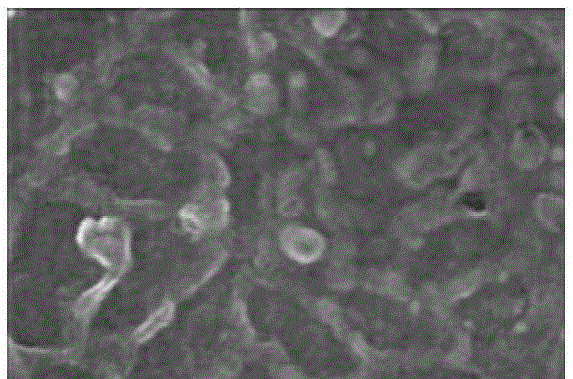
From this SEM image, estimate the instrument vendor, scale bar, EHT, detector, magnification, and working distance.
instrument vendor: Hitachi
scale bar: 500 nm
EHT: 5 kV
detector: SE(M)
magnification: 100 K X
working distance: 8.3 mm